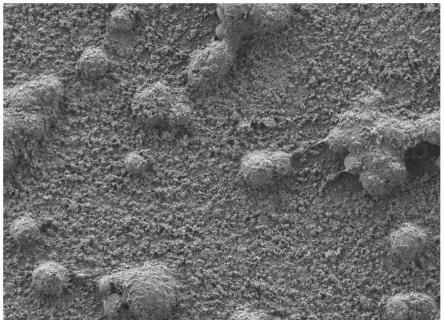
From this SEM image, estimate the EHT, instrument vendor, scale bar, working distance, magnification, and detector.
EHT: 2 kV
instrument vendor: JEOL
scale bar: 10000 nm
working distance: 4 mm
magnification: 1 K X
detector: LED